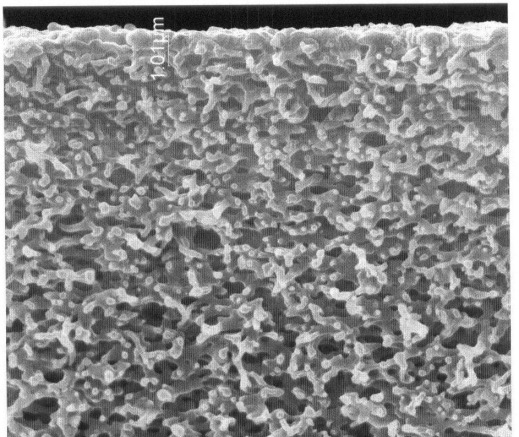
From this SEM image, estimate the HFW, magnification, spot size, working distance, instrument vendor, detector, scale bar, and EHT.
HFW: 14.9 µm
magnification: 20 K X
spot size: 2.5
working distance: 8.8 mm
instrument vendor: FEI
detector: ETD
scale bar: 5000 nm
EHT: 30 kV